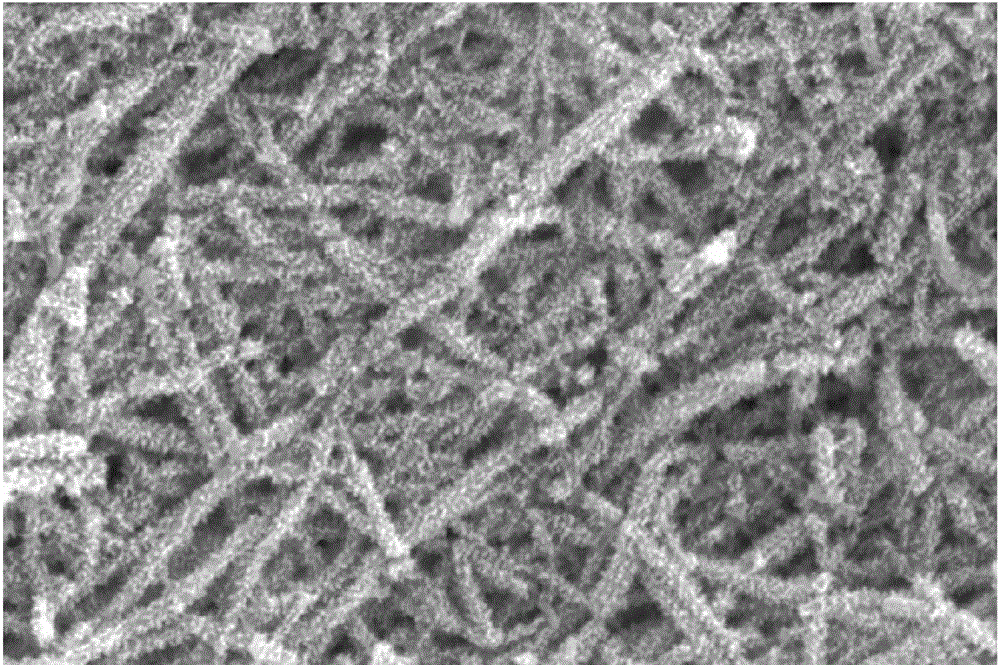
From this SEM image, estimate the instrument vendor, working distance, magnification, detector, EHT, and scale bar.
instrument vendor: Hitachi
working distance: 6.8 mm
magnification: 8 K X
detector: SE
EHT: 20 kV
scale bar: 5000 nm